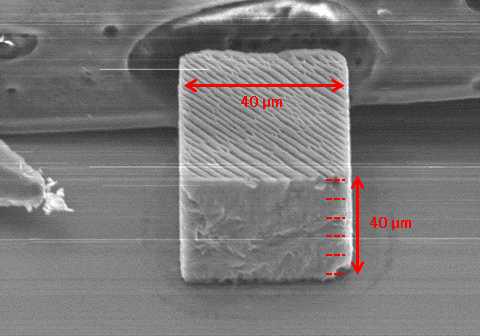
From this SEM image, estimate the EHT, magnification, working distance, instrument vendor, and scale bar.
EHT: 15 kV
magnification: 1.1 K X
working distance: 15.7 mm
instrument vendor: Hitachi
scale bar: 50000 nm